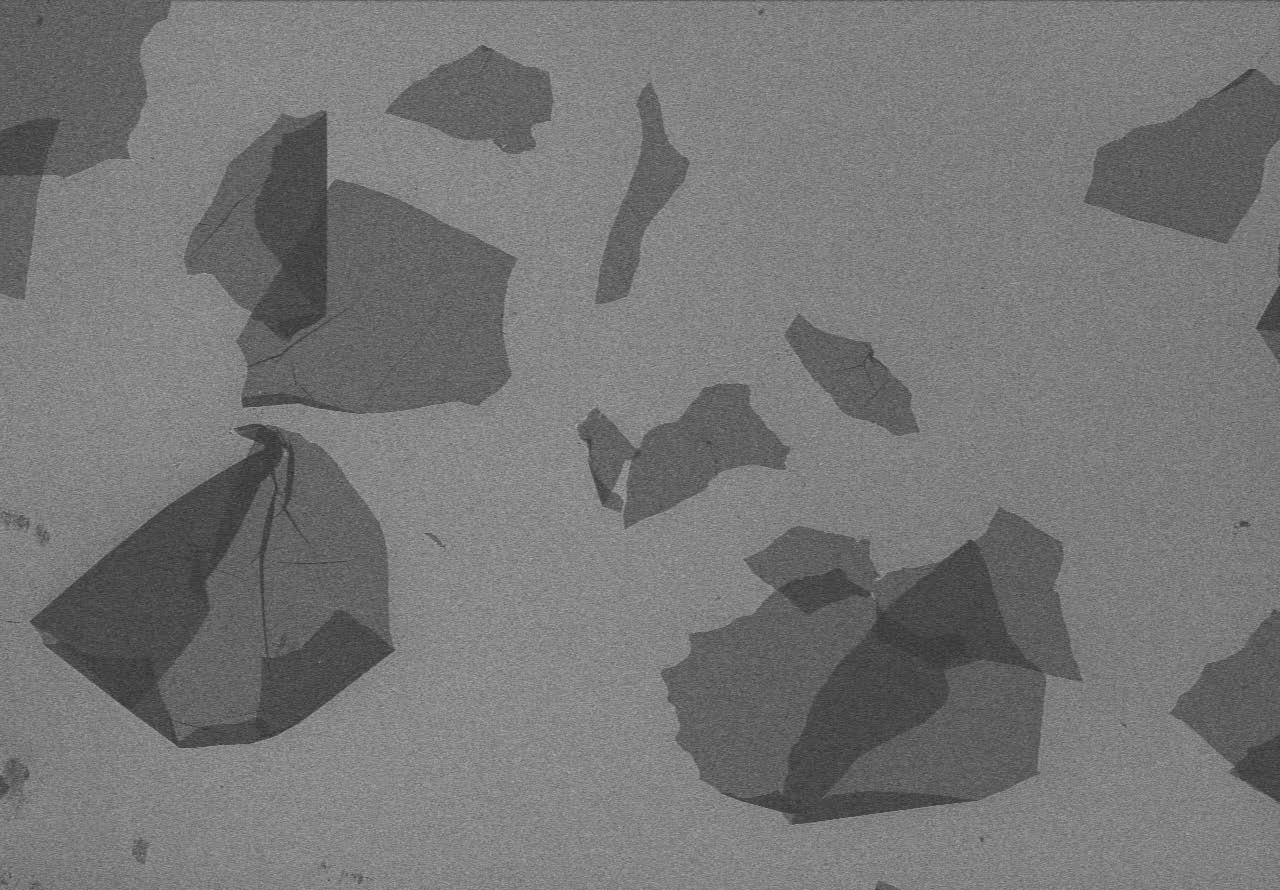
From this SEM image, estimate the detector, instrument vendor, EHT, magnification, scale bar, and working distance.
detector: SE(M)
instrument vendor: Hitachi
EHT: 3 kV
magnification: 0.3 K X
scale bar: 100000 nm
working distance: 10.8 mm